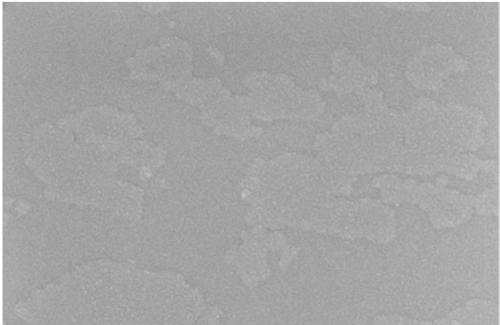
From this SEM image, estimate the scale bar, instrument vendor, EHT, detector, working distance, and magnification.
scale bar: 100 nm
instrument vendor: Zeiss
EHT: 3 kV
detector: SE2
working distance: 3.6 mm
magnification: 100 K X